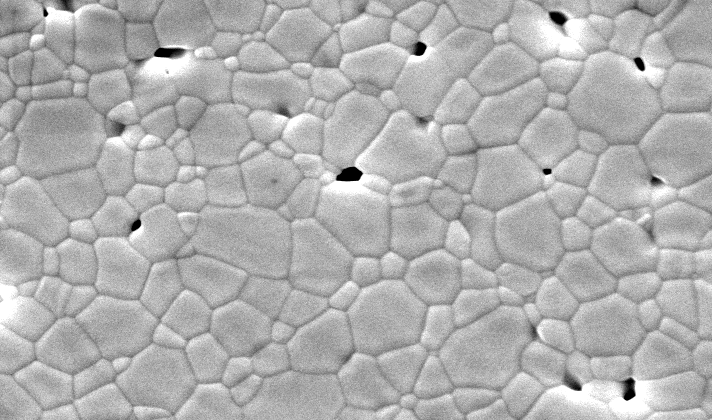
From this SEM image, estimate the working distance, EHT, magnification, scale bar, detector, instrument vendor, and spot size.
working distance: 10.5 mm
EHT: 20 kV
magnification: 8 K X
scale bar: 2000 nm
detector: SE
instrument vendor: FEI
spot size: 3.5